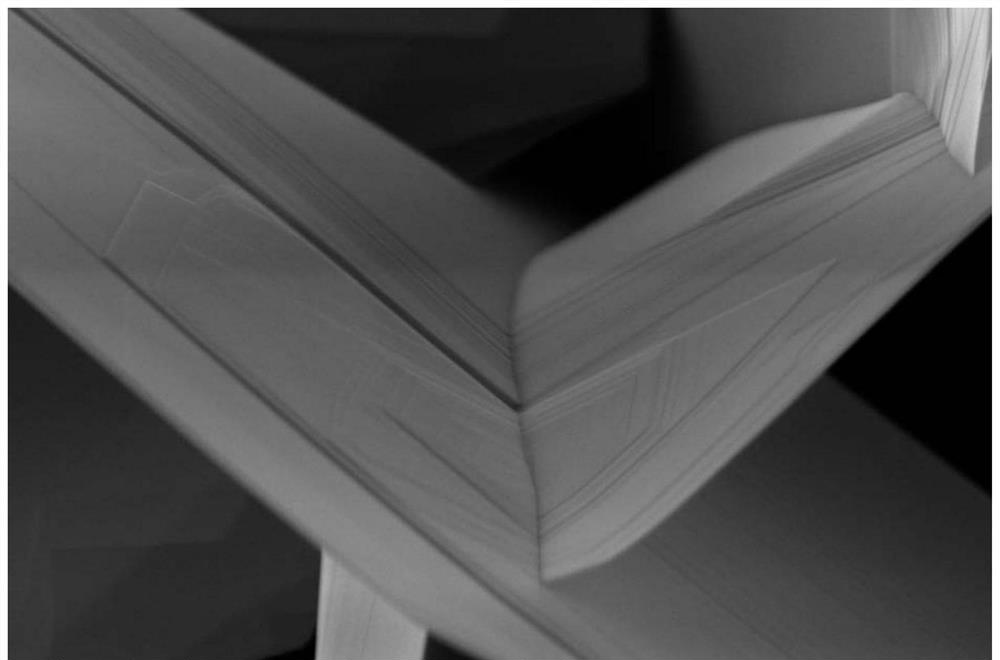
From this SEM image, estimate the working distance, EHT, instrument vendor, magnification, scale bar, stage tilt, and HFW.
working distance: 4.2 mm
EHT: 5 kV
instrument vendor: FEI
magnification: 50 K X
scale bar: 1000 nm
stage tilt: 0°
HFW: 5.12 µm